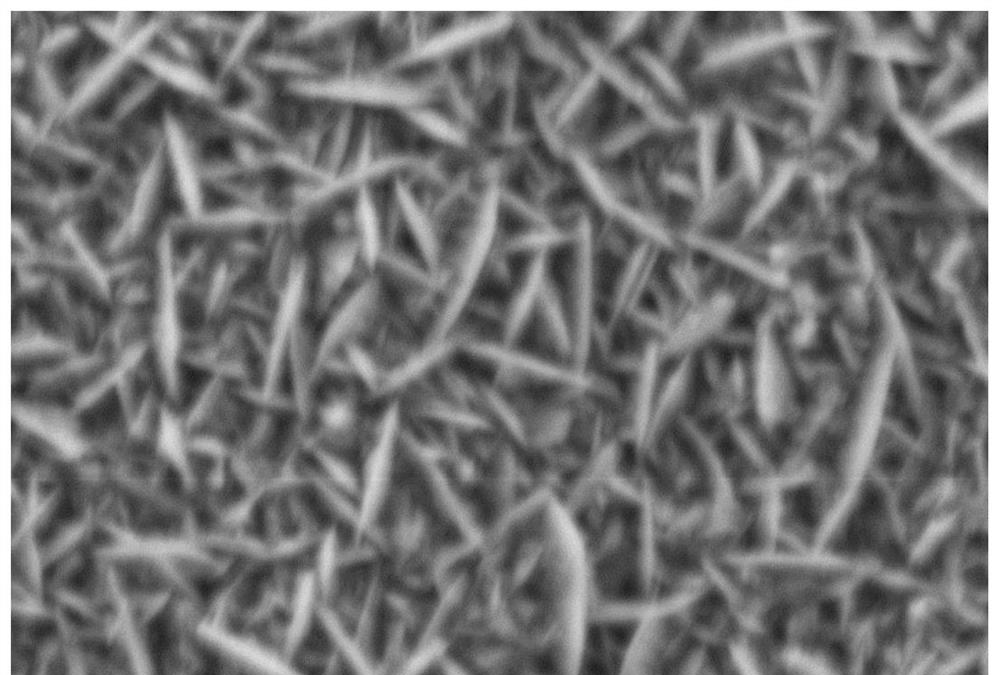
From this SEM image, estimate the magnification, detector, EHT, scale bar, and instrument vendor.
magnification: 5 K X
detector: SEI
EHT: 15 kV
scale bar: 5000 nm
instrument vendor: JEOL